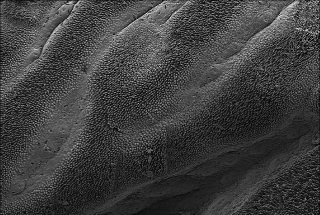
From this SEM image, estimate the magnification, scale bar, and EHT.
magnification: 0.1 K X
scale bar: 200000 nm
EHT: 5 kV